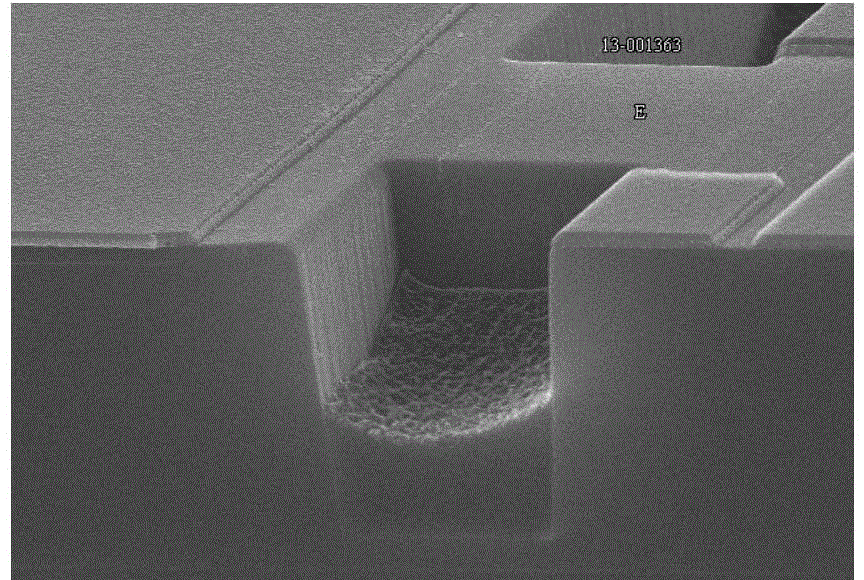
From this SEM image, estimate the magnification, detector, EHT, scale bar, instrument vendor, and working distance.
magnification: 15 K X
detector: SE(U)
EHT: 15 kV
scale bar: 3000 nm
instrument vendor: Hitachi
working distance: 7.5 mm